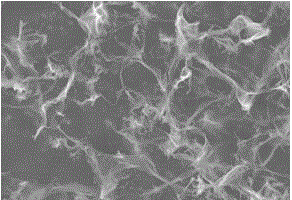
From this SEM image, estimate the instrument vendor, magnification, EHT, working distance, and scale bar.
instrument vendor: Hitachi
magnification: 10 K X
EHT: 10 kV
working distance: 11.2 mm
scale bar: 5000 nm